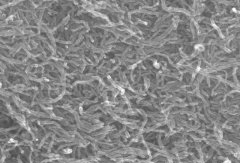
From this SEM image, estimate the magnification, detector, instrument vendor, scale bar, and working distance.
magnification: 50 K X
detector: SE(M)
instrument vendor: Hitachi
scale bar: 1000 nm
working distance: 13.1 mm